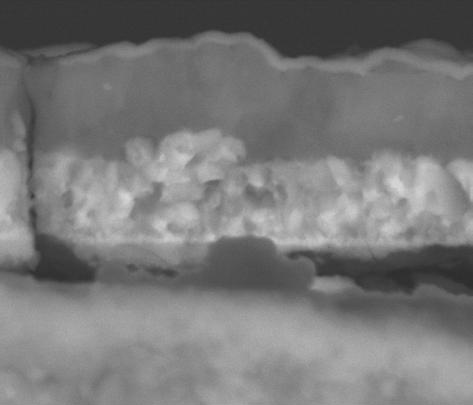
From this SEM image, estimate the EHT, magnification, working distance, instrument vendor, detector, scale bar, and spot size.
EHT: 30 kV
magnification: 6 K X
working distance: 11.4 mm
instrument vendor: FEI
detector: BSE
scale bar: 10000 nm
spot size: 3